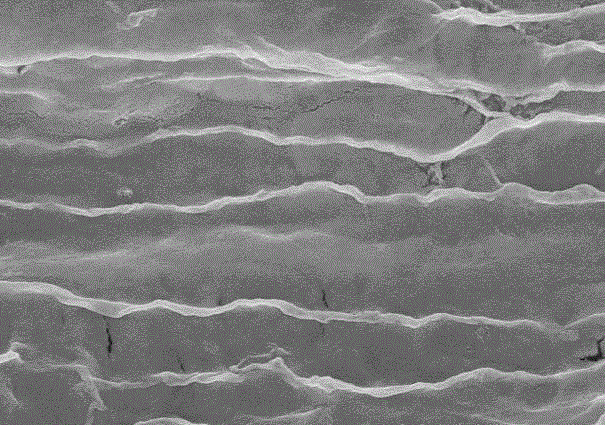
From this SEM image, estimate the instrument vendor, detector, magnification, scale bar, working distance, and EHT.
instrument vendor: Hitachi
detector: SE(U)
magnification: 20 K X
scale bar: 2000 nm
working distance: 11.6 mm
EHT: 10 kV